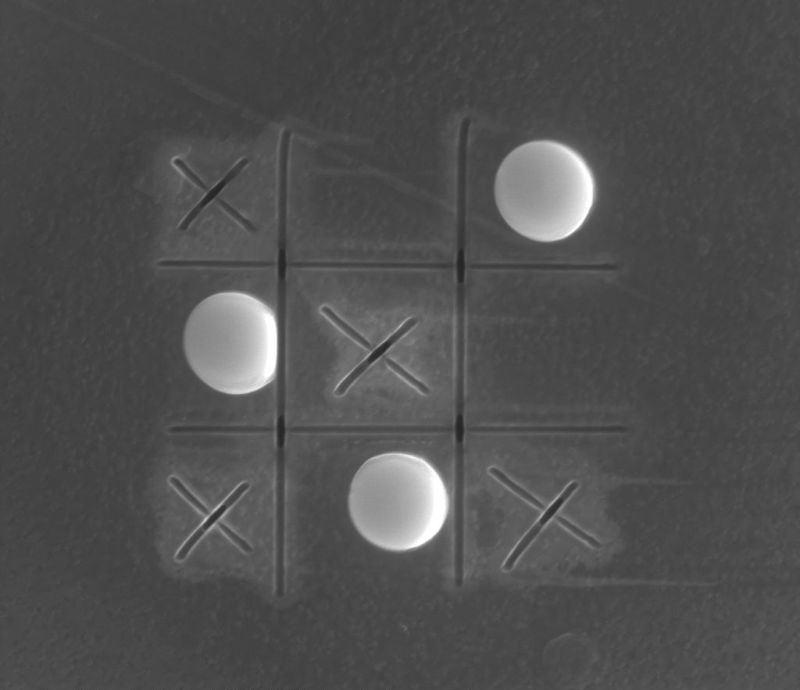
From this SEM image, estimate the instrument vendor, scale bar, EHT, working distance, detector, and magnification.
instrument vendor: FEI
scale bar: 4000 nm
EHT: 20 kV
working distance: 4 mm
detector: SE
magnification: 12.5 K X